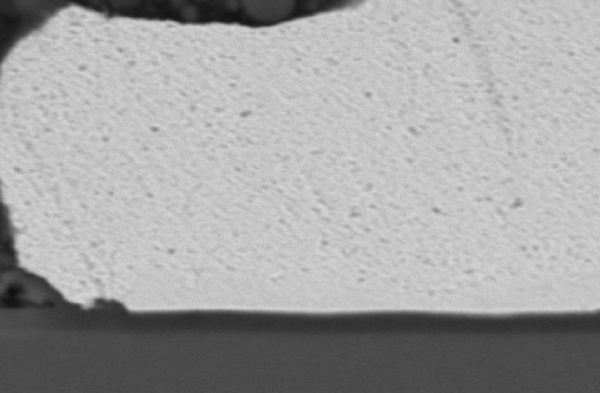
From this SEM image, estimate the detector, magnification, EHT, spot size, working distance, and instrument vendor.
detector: SEI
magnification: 3.7 K X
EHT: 15 kV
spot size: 48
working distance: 35 mm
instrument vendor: JEOL